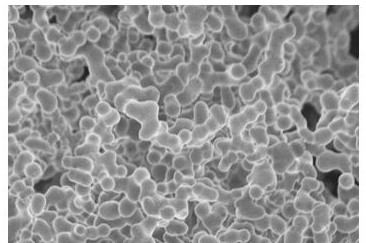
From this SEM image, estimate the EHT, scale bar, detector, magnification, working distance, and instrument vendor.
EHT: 3 kV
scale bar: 20000 nm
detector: SE(UL)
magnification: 2 K X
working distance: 9.2 mm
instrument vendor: Hitachi